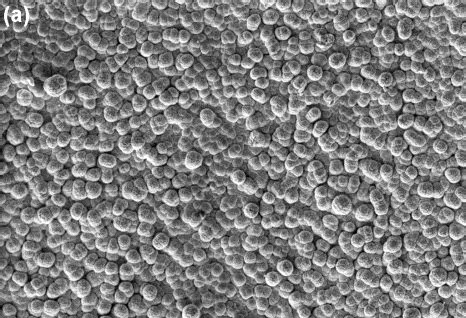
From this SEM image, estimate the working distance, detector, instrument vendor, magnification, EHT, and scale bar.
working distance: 14.1 mm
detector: SE2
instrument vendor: Zeiss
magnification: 0.5 K X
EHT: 15 kV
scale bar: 20000 nm